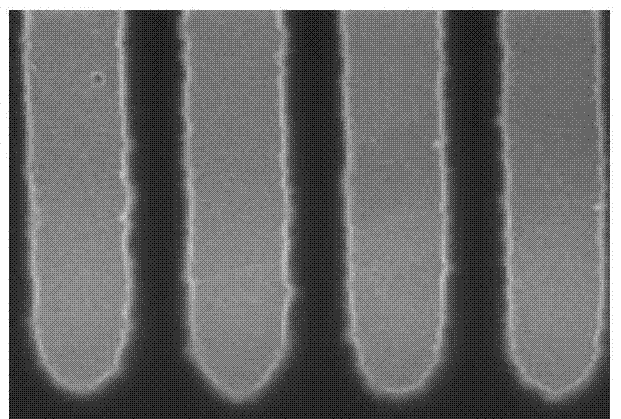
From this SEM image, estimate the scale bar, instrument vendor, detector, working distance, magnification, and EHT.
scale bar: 500 nm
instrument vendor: Hitachi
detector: SE(U)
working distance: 9.5 mm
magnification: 100 K X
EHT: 15 kV